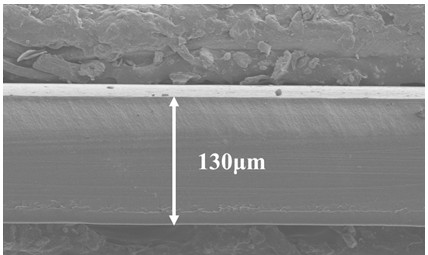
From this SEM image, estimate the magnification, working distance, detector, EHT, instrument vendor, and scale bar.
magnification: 0.3 K X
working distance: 8.4 mm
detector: LM(UL)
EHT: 5 kV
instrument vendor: Hitachi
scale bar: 100000 nm